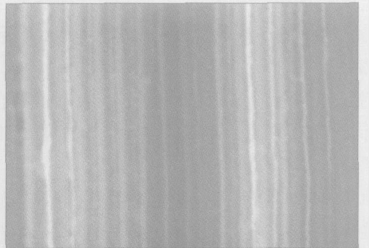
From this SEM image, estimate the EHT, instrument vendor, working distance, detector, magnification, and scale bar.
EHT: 10 kV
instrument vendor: Hitachi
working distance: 7.9 mm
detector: SE(L)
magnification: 70 K X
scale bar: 500 nm